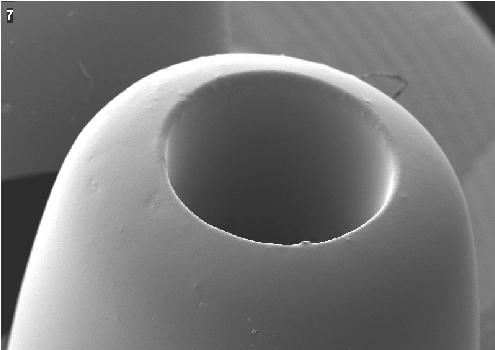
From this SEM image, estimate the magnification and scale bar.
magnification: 0.051 K X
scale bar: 500000 nm